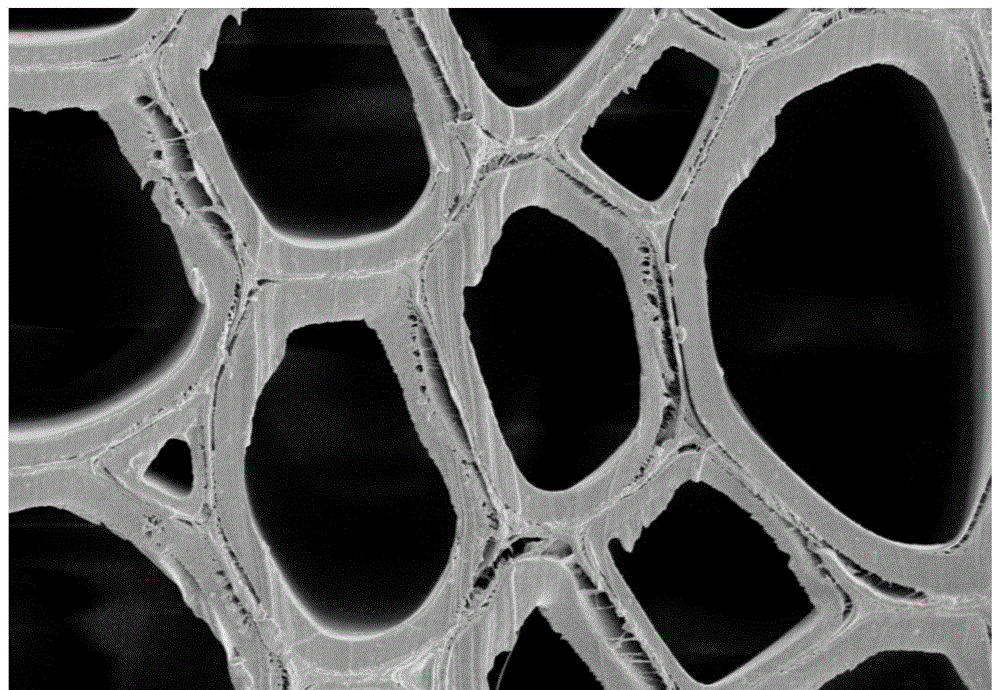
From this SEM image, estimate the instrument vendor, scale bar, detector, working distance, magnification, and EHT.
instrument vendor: Hitachi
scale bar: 20000 nm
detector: SE(TUL)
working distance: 8.6 mm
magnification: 2 K X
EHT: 3 kV